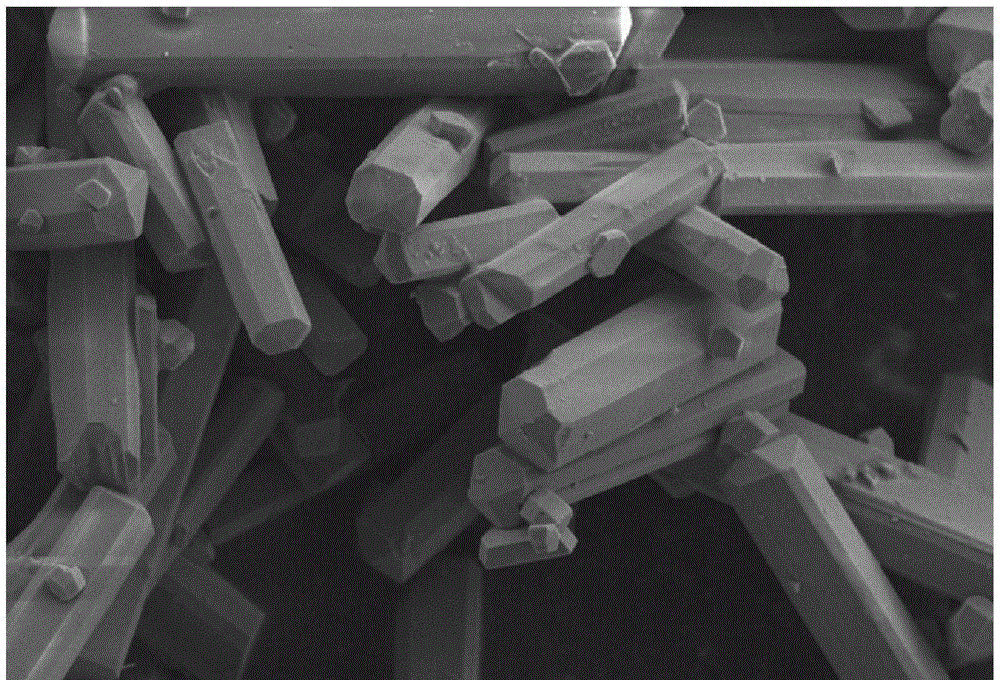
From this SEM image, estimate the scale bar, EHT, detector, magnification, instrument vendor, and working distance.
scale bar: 2000 nm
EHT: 3 kV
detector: SE2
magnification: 10 K X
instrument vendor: Zeiss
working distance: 5.7 mm